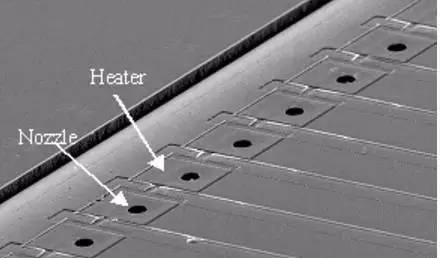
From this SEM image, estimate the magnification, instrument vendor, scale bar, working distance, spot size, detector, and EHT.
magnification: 0.1 K X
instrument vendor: FEI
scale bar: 200000 nm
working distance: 22.8 mm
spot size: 3.1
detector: SE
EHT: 20 kV